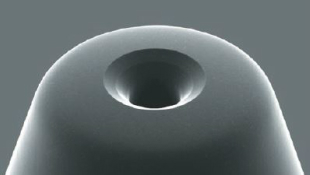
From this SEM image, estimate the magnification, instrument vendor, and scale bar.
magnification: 0.5 K X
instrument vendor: JEOL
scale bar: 50000 nm